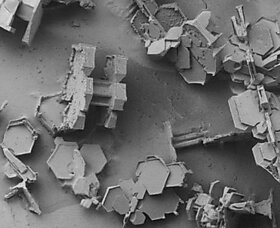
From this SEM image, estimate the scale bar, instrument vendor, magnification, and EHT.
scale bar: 1000000 nm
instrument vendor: JEOL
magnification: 0.03 K X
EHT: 2 kV